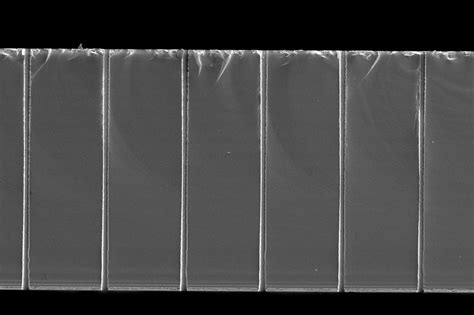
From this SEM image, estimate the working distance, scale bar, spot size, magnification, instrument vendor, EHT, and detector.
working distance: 26 mm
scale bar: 100000 nm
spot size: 51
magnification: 0.22 K X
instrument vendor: JEOL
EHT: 5 kV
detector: SEI